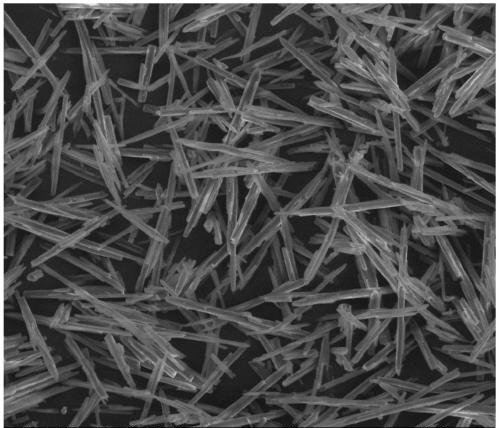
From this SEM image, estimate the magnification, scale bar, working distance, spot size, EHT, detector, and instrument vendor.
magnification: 0.2 K X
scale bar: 100000 nm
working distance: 10.4 mm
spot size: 3.5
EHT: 20 kV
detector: LFD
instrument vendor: FEI